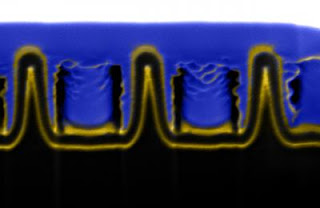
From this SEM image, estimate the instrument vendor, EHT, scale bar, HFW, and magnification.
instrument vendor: other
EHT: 30 kV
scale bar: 500 nm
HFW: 4.27 µm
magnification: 30 K X